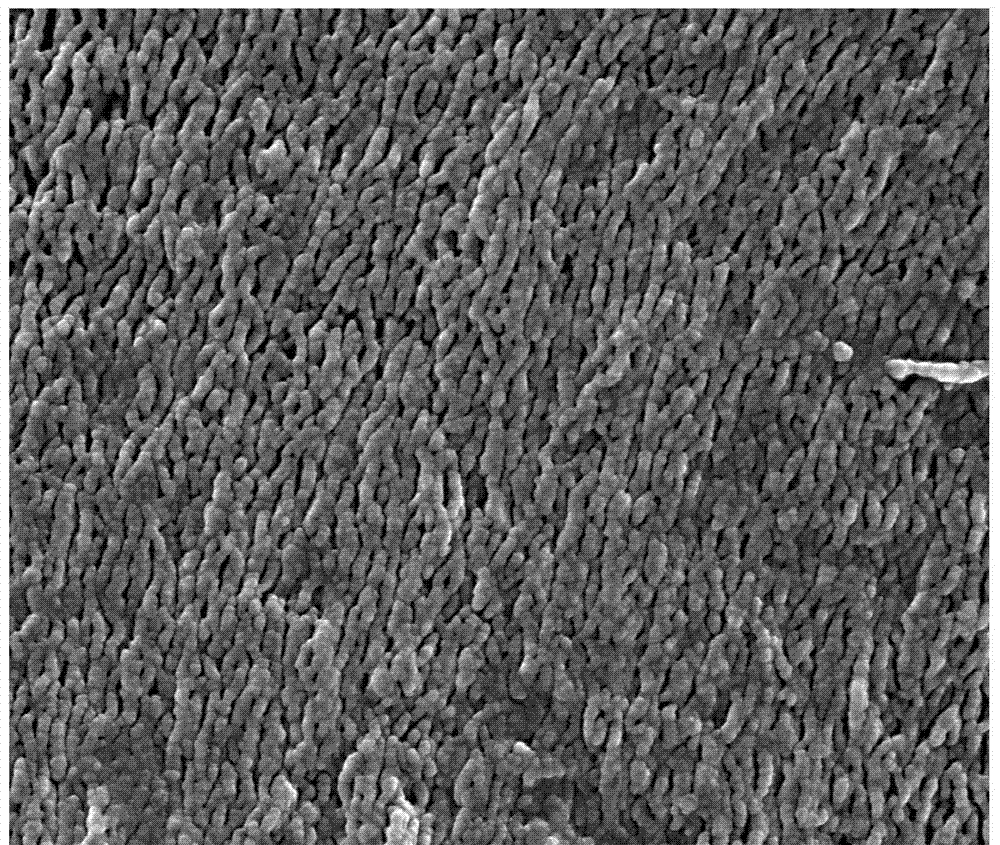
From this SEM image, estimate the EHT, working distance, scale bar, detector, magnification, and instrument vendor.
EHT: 20 kV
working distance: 10.7 mm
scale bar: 1000 nm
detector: SE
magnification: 80 K X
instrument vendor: FEI